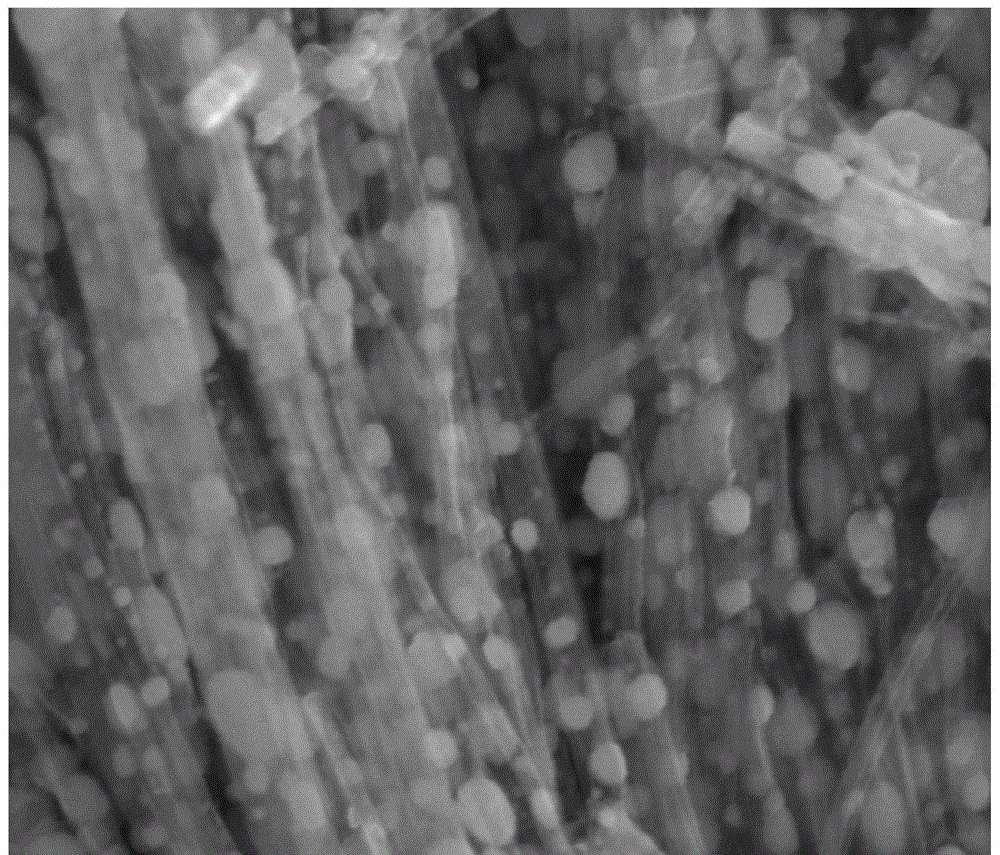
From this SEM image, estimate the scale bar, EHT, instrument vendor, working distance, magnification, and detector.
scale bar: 500 nm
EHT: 10 kV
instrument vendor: FEI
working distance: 5.1 mm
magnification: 160 K X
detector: TLD